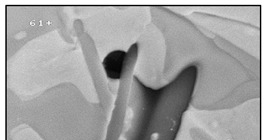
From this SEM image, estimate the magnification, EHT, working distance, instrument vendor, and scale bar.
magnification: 4.5 K X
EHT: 20 kV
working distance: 14 mm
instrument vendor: JEOL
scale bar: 1000 nm